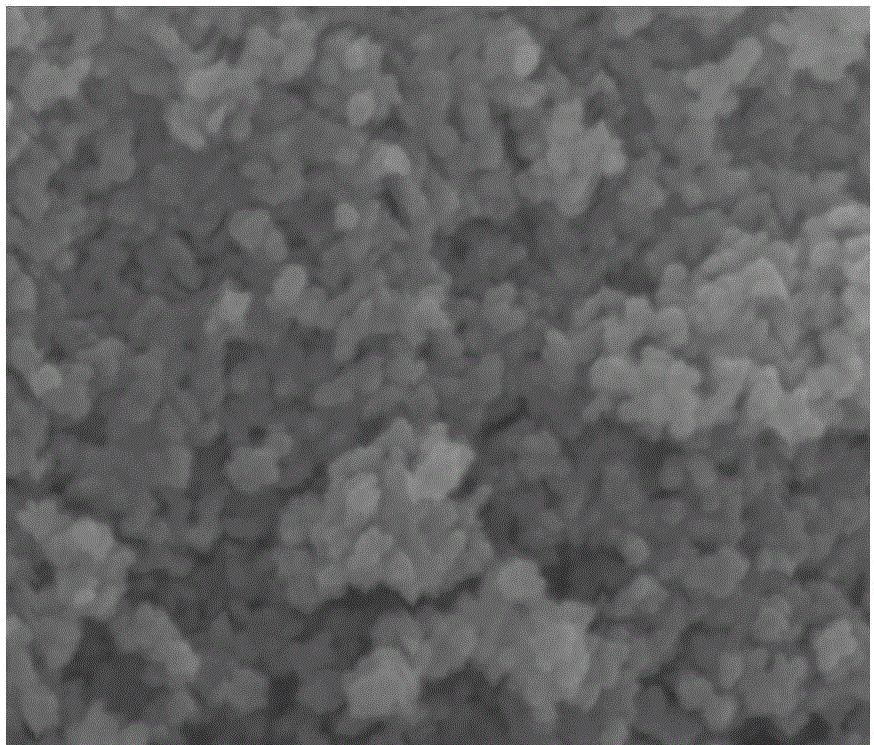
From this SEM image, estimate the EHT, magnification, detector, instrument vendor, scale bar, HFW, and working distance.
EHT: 10 kV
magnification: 200 K X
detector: TLD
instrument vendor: FEI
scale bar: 400 nm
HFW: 1.2 µm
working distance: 5 mm